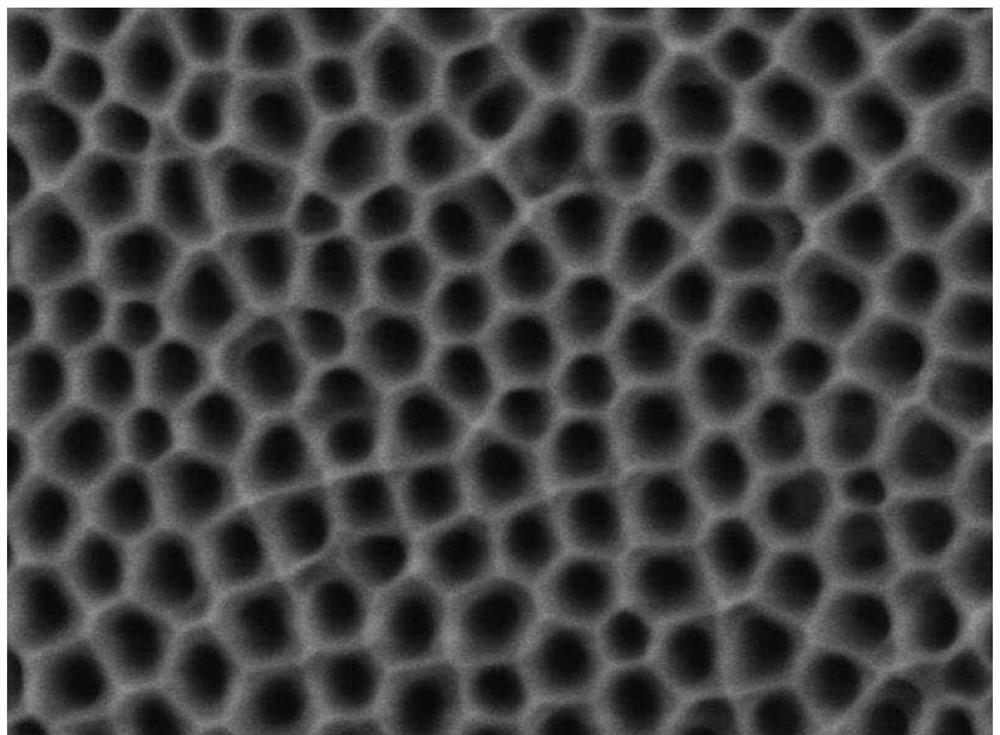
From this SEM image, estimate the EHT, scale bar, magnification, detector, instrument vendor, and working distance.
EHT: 3 kV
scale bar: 1000 nm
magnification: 22 K X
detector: LED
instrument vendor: JEOL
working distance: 11 mm